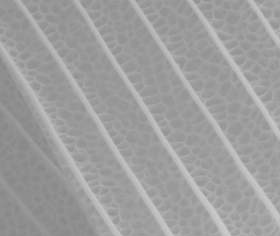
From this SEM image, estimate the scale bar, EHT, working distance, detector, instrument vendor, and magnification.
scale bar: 5000 nm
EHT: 20 kV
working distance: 10.9 mm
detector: LFD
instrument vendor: FEI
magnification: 15 K X